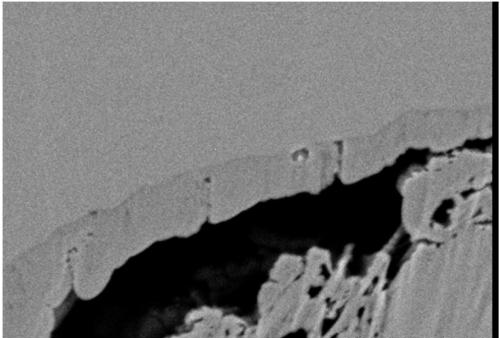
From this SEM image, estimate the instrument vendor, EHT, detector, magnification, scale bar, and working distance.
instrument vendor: Hitachi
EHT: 1 kV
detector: LA100(U)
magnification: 70 K X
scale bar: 500 nm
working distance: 2.4 mm